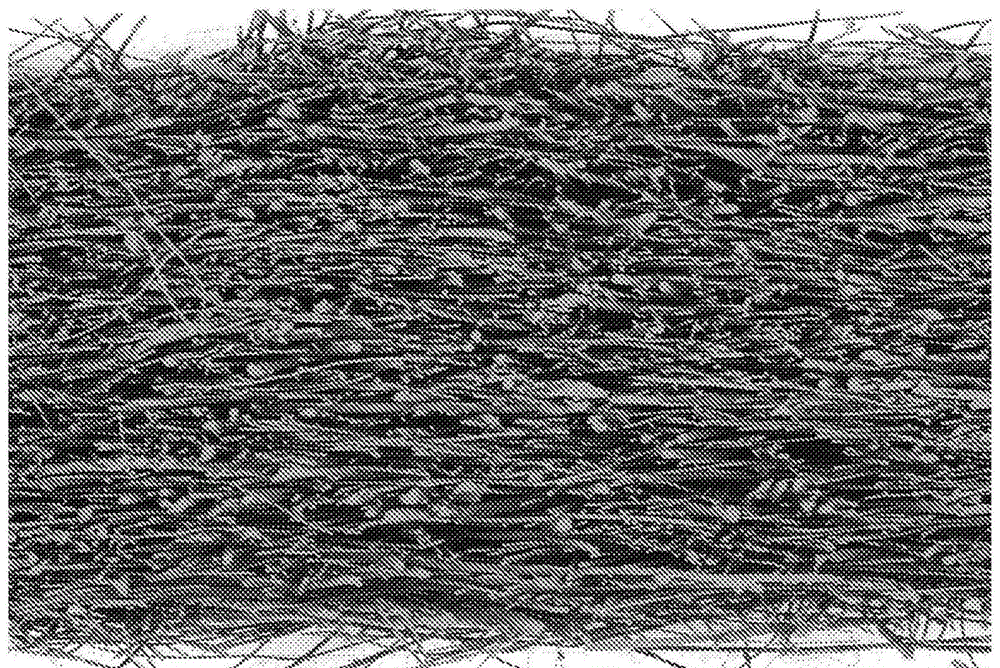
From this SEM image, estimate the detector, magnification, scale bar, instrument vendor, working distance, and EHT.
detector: NTS BSD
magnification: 0.046 K X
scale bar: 200000 nm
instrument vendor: Zeiss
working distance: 10.5 mm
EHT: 15 kV